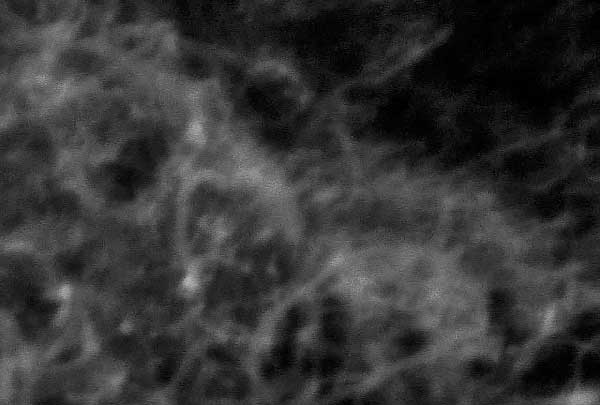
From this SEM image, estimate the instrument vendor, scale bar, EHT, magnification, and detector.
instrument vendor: JEOL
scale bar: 500 nm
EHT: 20 kV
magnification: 30 K X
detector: SEI